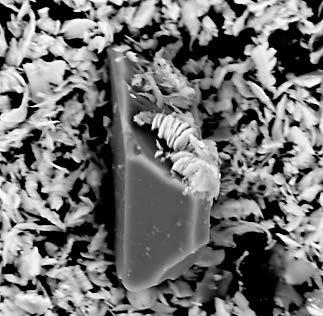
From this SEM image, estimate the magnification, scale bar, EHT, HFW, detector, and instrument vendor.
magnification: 9.3 K X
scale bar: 8000 nm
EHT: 15 kV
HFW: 29.1 µm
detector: BSD Full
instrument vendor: other